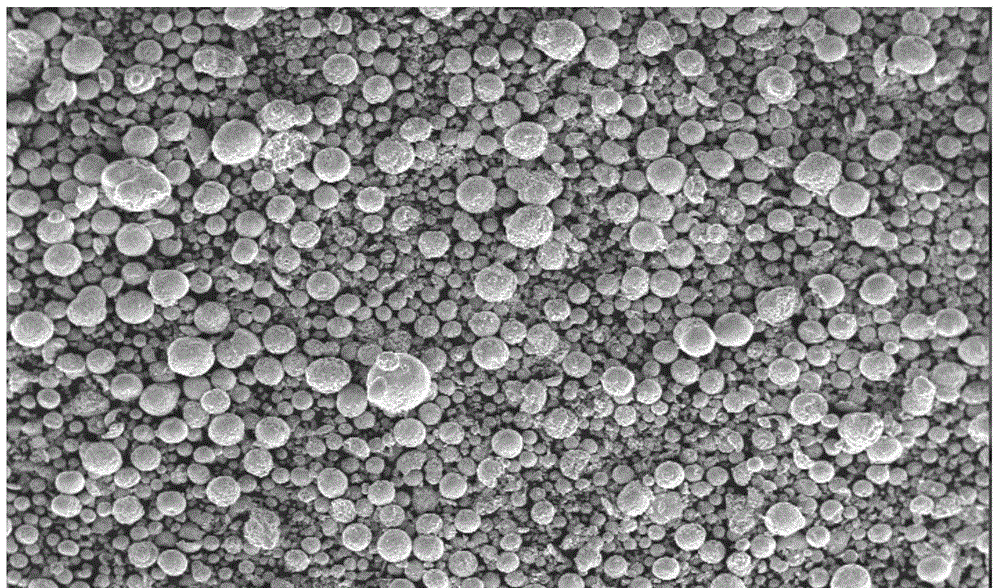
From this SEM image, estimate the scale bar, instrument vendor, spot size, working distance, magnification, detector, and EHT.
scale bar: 500000 nm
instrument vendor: FEI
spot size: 3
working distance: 10.3 mm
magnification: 0.05 K X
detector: SE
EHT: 5 kV